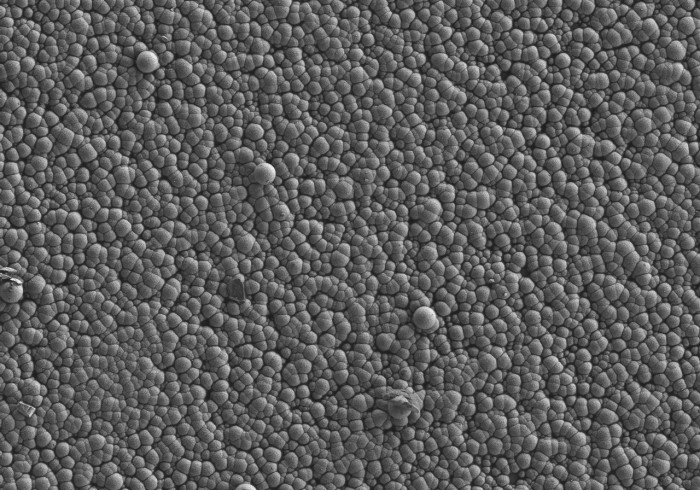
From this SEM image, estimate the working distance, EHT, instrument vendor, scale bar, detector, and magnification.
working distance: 9.2 mm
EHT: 2 kV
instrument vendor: Hitachi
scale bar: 50000 nm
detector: SE(L)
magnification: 1 K X